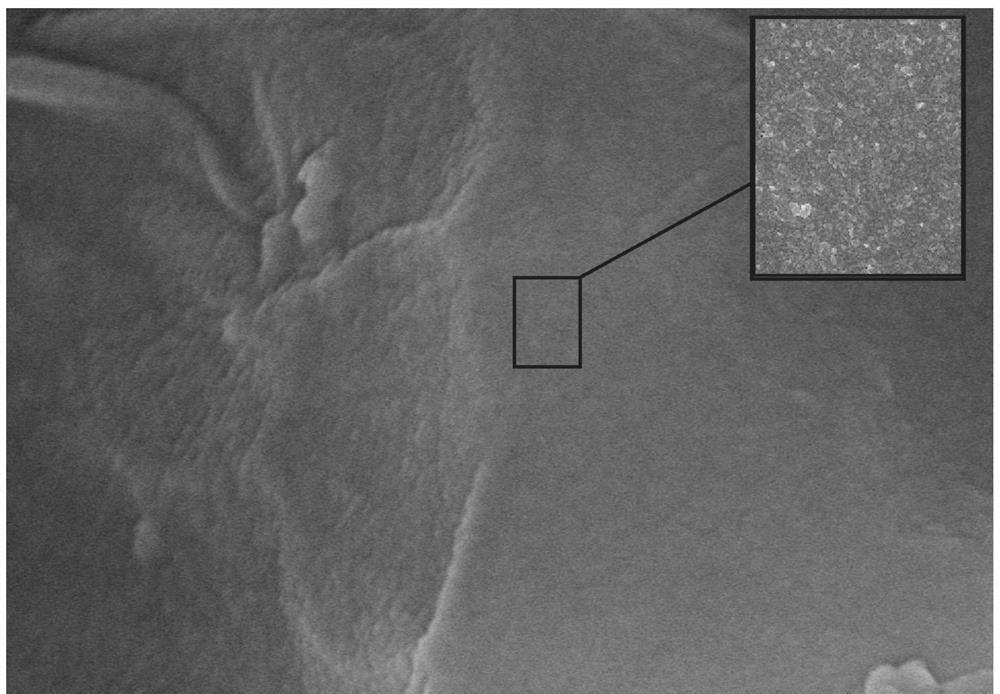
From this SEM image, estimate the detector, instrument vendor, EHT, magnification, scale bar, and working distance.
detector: SE(U)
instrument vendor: Hitachi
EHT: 15 kV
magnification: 100 K X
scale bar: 500 nm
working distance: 6 mm